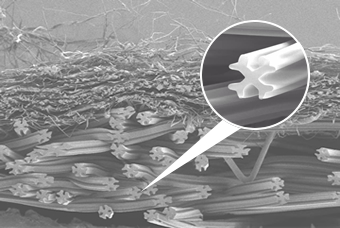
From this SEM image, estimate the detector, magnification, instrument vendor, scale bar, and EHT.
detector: SEI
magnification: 0.1 K X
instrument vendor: JEOL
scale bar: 100000 nm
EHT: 3 kV